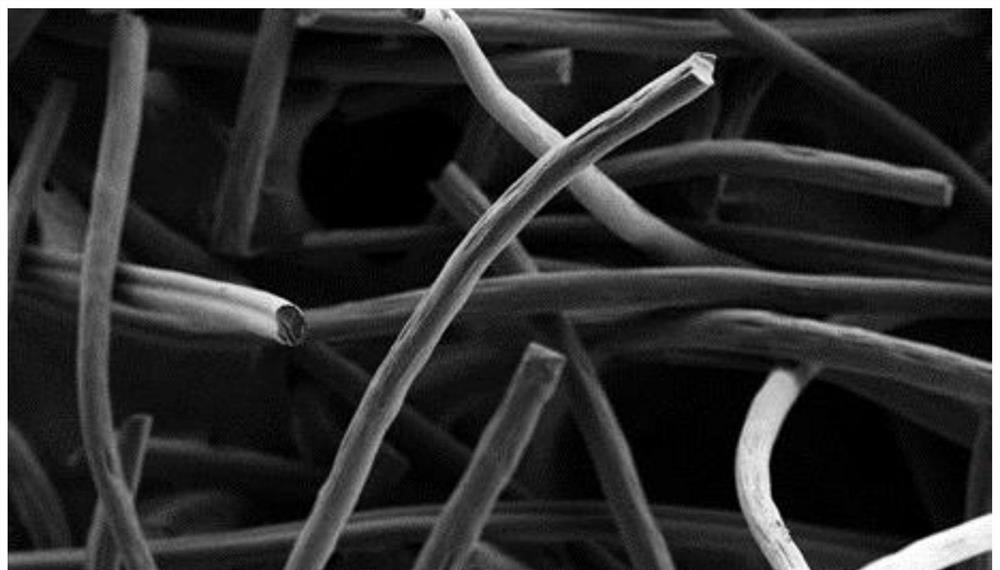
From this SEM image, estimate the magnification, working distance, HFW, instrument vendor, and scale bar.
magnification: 0.5 K X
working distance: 2.78 mm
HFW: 558 µm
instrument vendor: Phenom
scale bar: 100000 nm